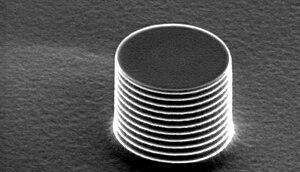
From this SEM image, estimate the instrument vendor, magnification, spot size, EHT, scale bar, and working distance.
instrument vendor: FEI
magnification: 5 K X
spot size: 3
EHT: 5 kV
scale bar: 5000 nm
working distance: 21.8 mm